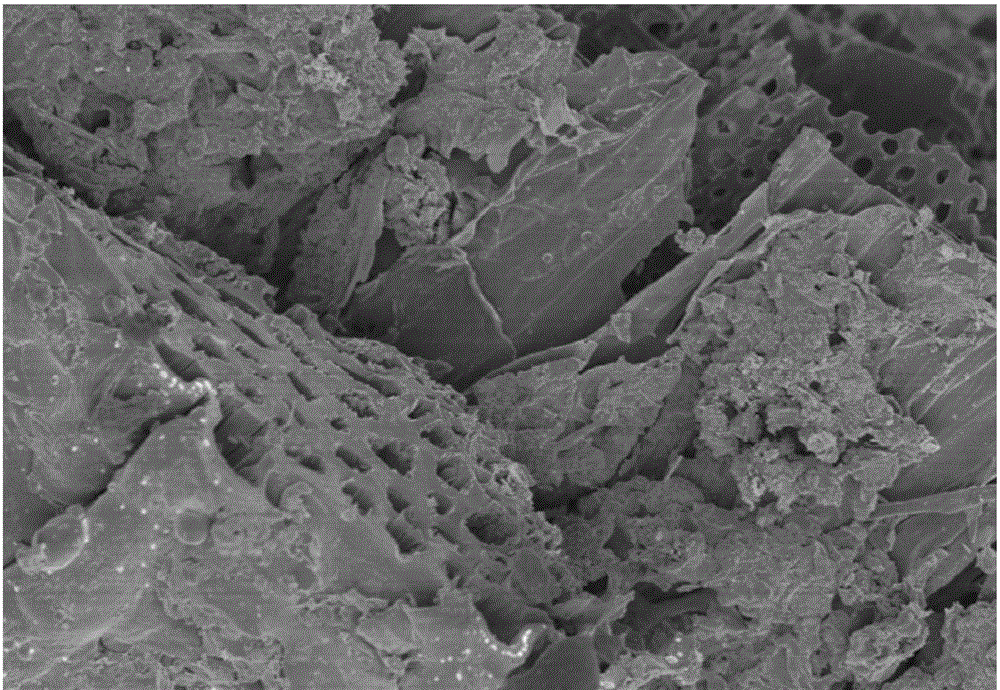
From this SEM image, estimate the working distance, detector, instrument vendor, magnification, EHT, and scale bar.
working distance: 10.1 mm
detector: SE(UL)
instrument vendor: Hitachi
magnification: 2.2 K X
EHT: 3 kV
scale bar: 20000 nm